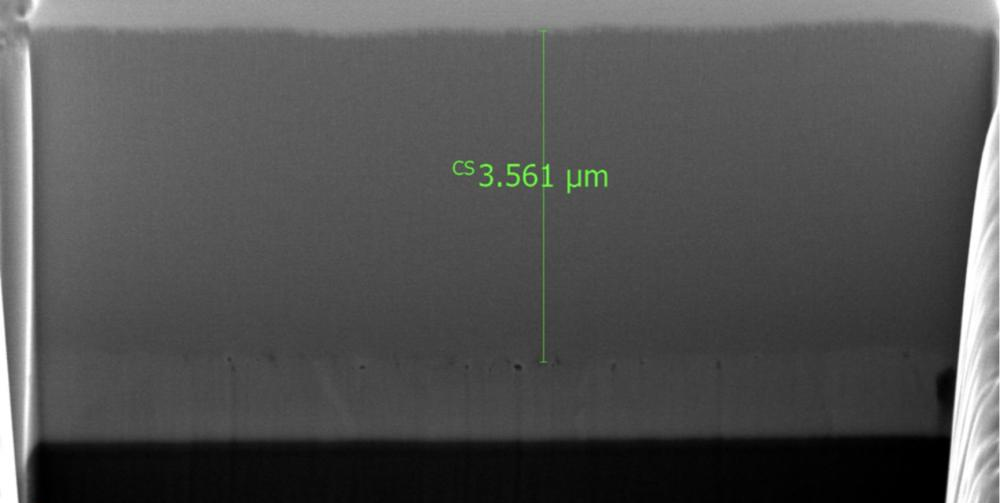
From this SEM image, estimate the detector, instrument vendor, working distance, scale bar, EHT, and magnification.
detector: TLD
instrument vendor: FEI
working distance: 4 mm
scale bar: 2000 nm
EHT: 10 kV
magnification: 15 K X